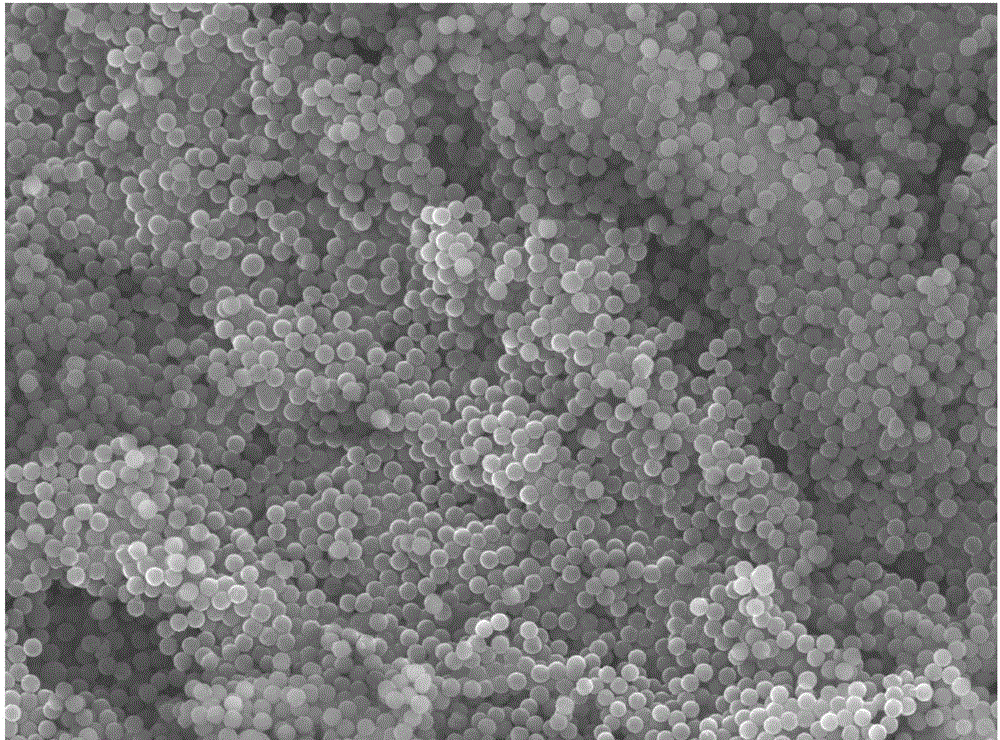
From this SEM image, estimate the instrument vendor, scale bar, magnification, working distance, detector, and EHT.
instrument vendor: JEOL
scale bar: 1000 nm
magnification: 5 K X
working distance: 12 mm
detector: LED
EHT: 15 kV